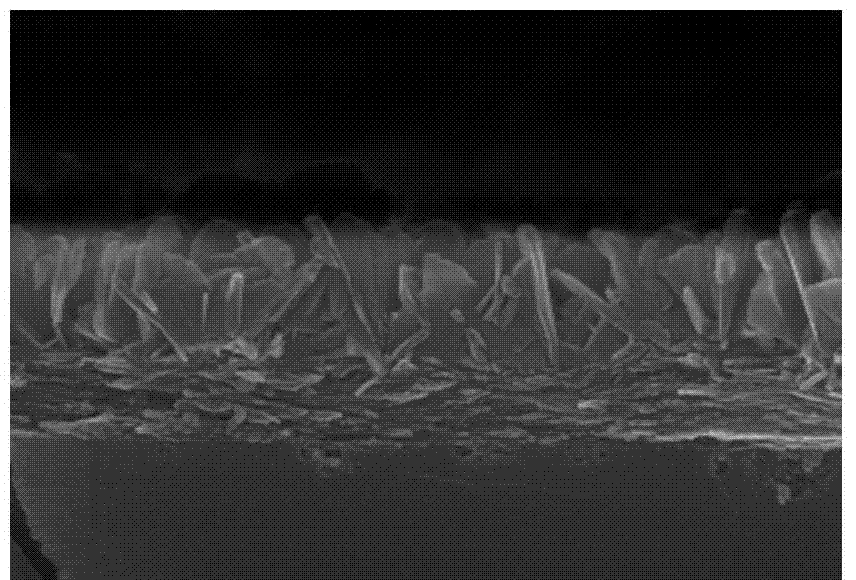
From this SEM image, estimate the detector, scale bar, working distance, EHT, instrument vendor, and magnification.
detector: SE(U)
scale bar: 1000 nm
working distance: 10.2 mm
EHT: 20 kV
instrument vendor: Hitachi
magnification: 50 K X